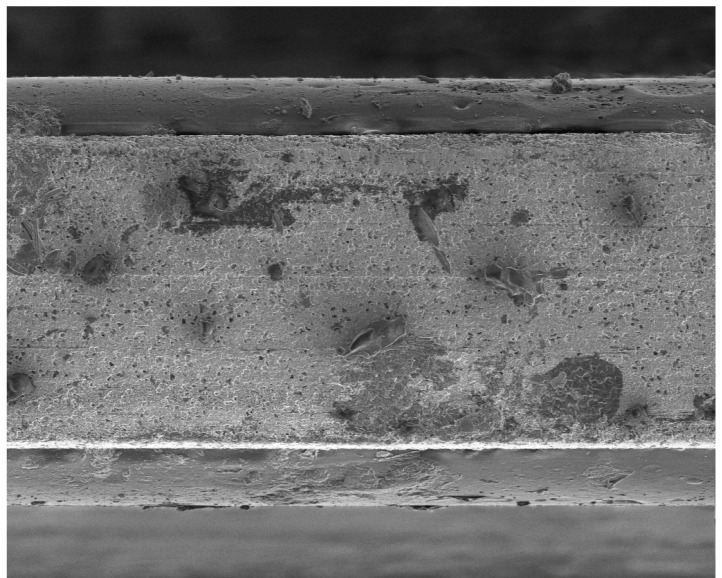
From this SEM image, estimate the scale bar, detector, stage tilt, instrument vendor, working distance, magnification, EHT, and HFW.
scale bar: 100000 nm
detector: SE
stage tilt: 0°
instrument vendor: FEI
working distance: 9.8 mm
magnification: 0.496 K X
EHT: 3 kV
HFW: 301 µm